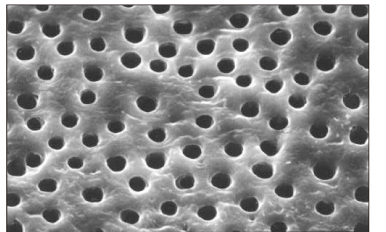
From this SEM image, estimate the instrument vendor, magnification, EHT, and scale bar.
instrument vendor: Hitachi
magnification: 2 K X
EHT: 20 kV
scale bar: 20000 nm